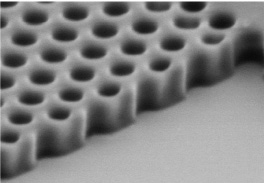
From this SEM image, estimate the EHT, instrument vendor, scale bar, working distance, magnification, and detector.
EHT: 2 kV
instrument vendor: Hitachi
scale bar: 1000 nm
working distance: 23 mm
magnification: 50 K X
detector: SE(M)